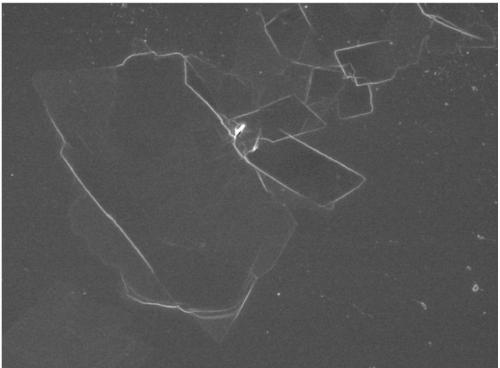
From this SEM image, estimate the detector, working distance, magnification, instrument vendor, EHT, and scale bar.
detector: SEI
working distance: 8.4 mm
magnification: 2.7 K X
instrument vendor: JEOL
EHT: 5 kV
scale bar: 10000 nm